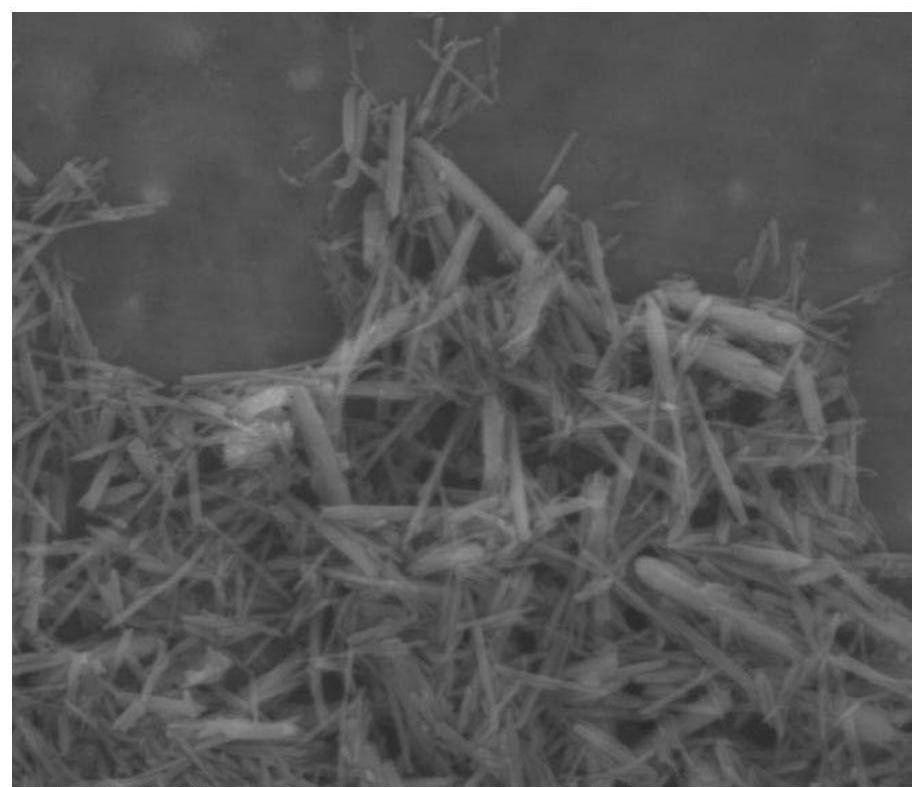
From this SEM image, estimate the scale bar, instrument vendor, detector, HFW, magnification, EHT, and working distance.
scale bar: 4000 nm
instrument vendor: FEI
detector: ETD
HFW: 15 µm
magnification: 20 K X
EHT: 10 kV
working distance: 5.2 mm